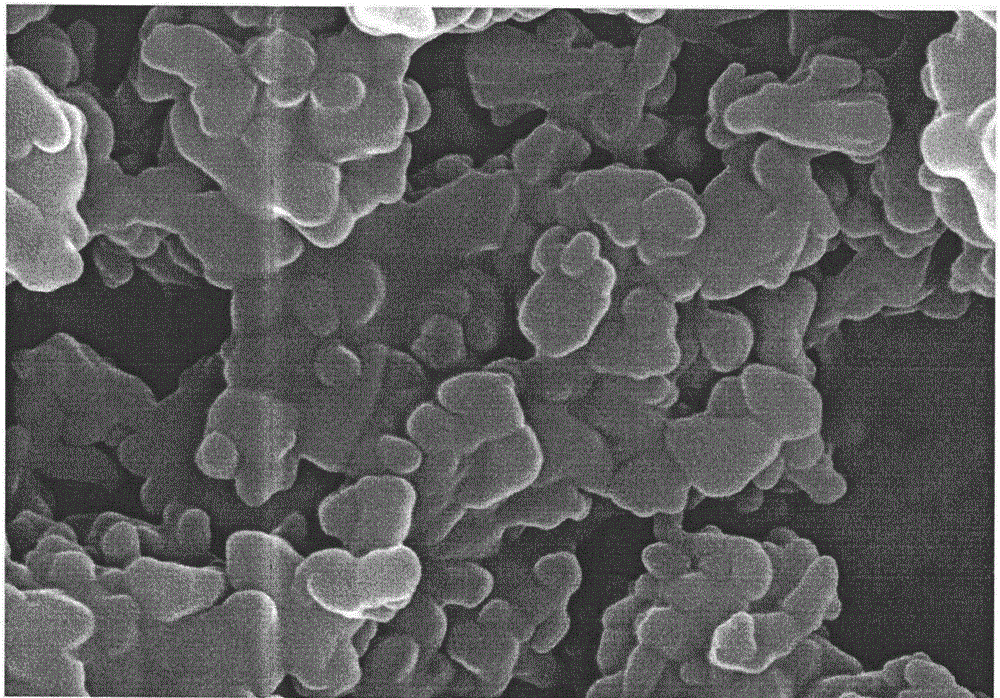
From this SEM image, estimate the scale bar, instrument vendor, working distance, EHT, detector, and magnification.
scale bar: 100 nm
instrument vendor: Zeiss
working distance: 7.1 mm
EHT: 10 kV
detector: InlensDuo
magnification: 50 K X